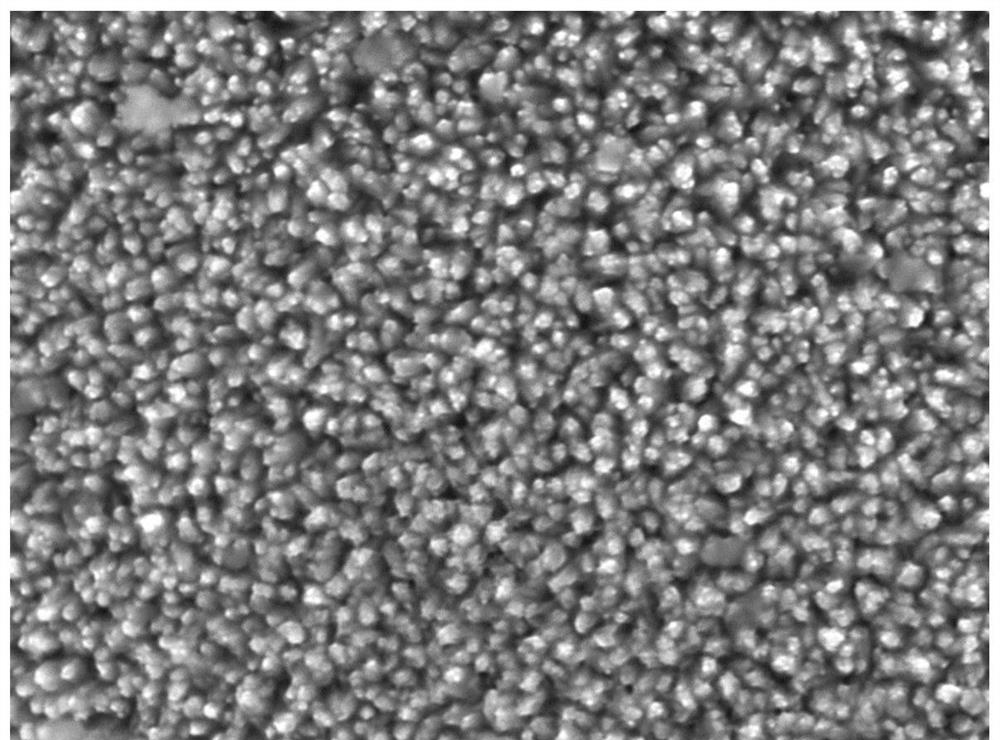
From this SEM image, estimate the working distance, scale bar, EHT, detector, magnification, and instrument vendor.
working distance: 20.7 mm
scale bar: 1000 nm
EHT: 15 kV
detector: LED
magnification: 10 K X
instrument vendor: JEOL